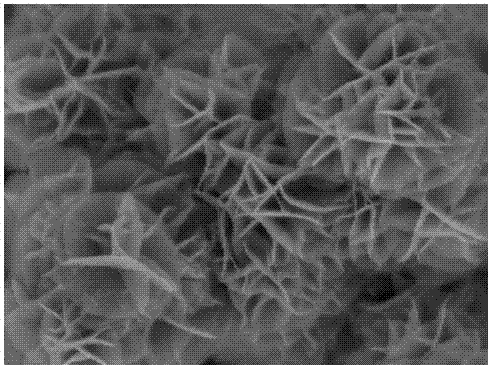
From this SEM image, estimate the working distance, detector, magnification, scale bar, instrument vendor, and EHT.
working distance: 7.3 mm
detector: InBeam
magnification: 100 K X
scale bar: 500 nm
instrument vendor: Tescan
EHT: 5 kV